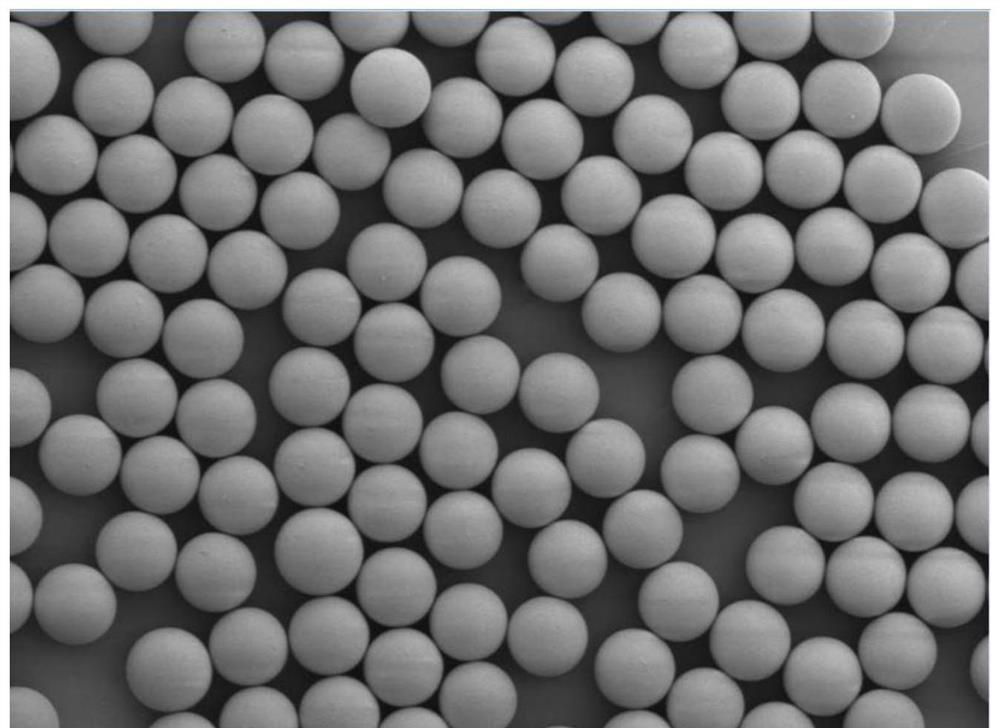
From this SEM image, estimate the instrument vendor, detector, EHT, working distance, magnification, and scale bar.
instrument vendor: JEOL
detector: LED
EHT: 2 kV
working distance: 10.1 mm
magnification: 0.6 K X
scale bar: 10000 nm